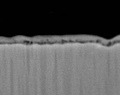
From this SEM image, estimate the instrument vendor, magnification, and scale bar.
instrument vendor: JEOL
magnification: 10 K X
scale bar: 1000 nm